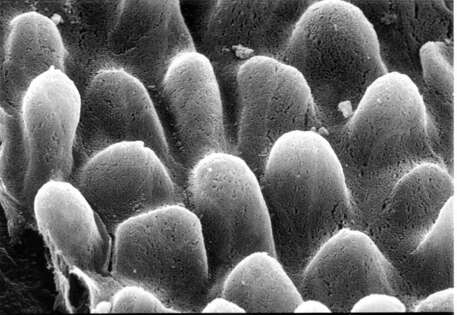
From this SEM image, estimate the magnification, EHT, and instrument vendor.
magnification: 2 K X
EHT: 20 kV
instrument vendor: JEOL